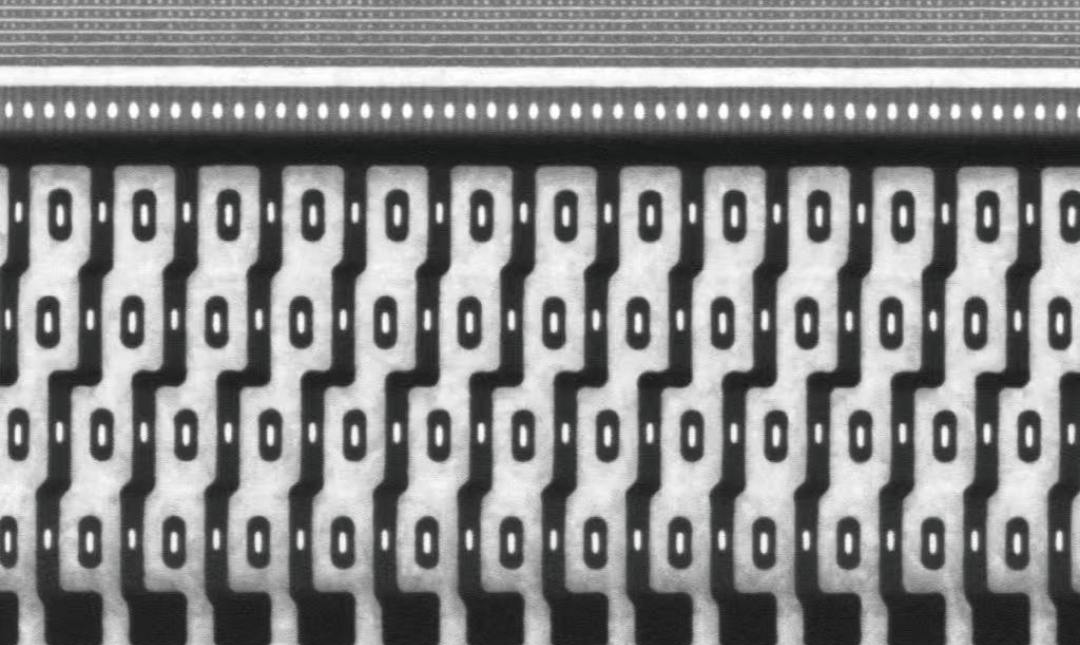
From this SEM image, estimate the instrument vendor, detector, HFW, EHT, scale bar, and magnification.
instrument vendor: other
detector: BSD Full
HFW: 5.37 µm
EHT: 15 kV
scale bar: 1000 nm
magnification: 50 K X